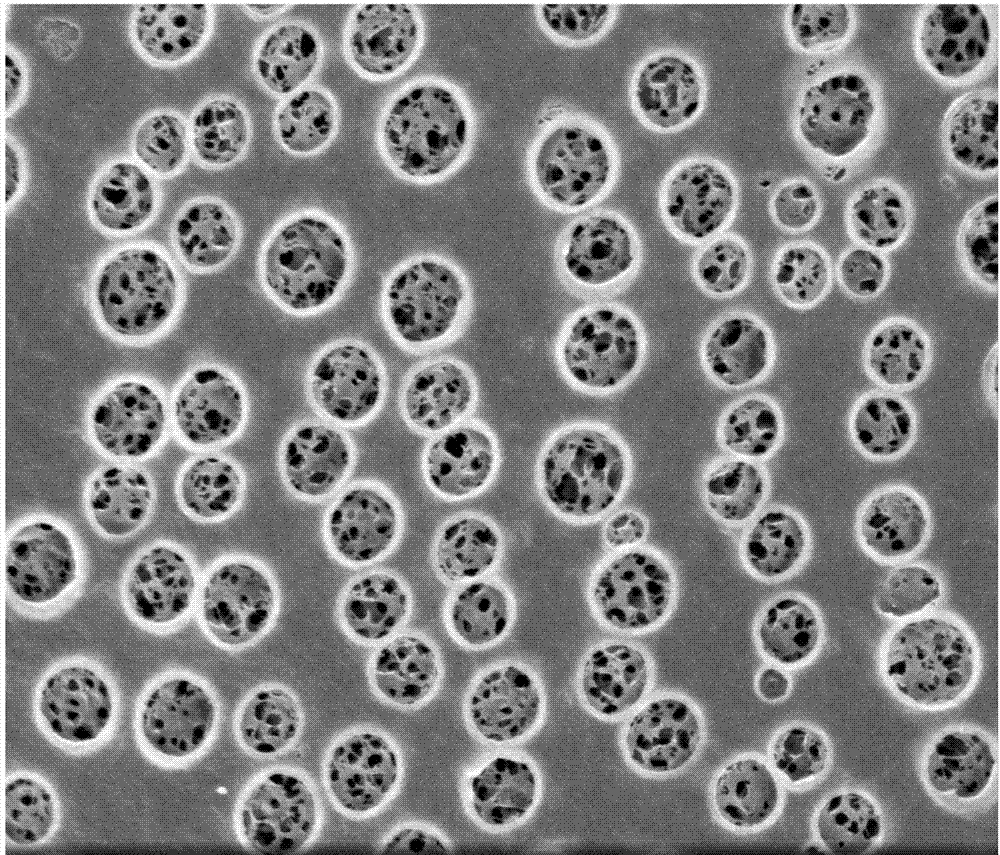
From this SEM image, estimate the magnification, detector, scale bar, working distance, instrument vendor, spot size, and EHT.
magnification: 10 K X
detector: TLD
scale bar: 5000 nm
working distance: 5.3 mm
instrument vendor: FEI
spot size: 3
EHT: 3 kV